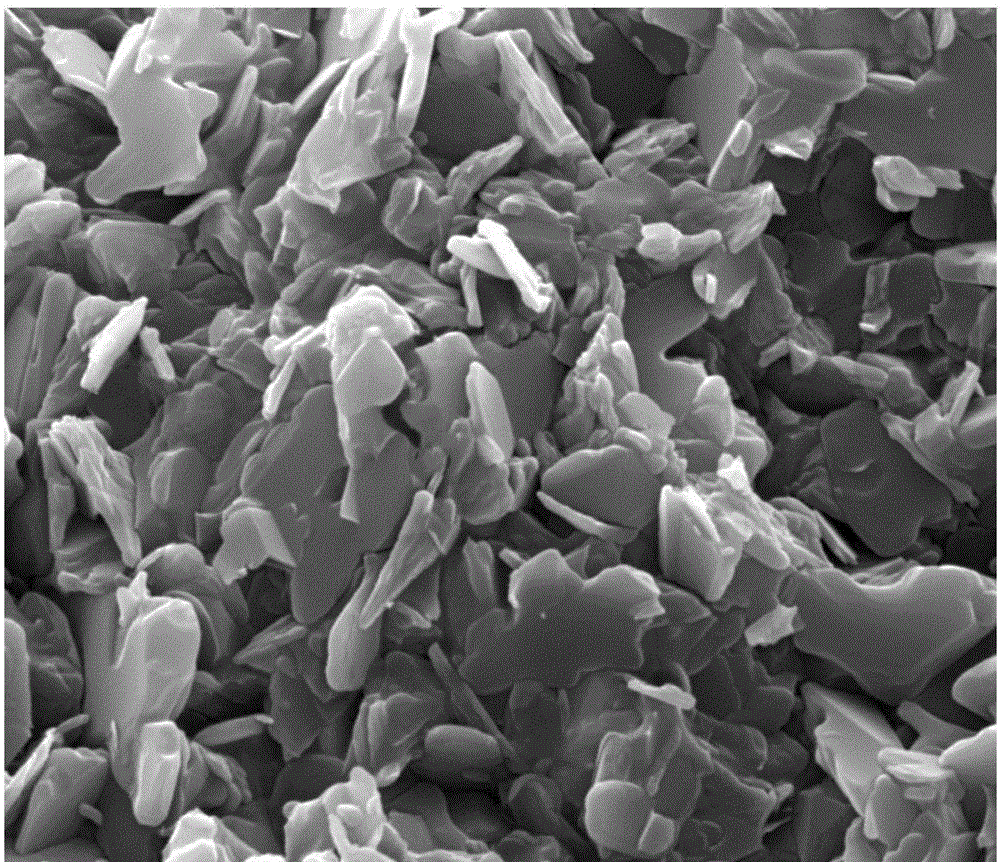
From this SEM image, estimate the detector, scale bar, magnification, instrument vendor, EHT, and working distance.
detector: SE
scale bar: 5000 nm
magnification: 20 K X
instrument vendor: FEI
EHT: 20 kV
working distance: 12.9 mm